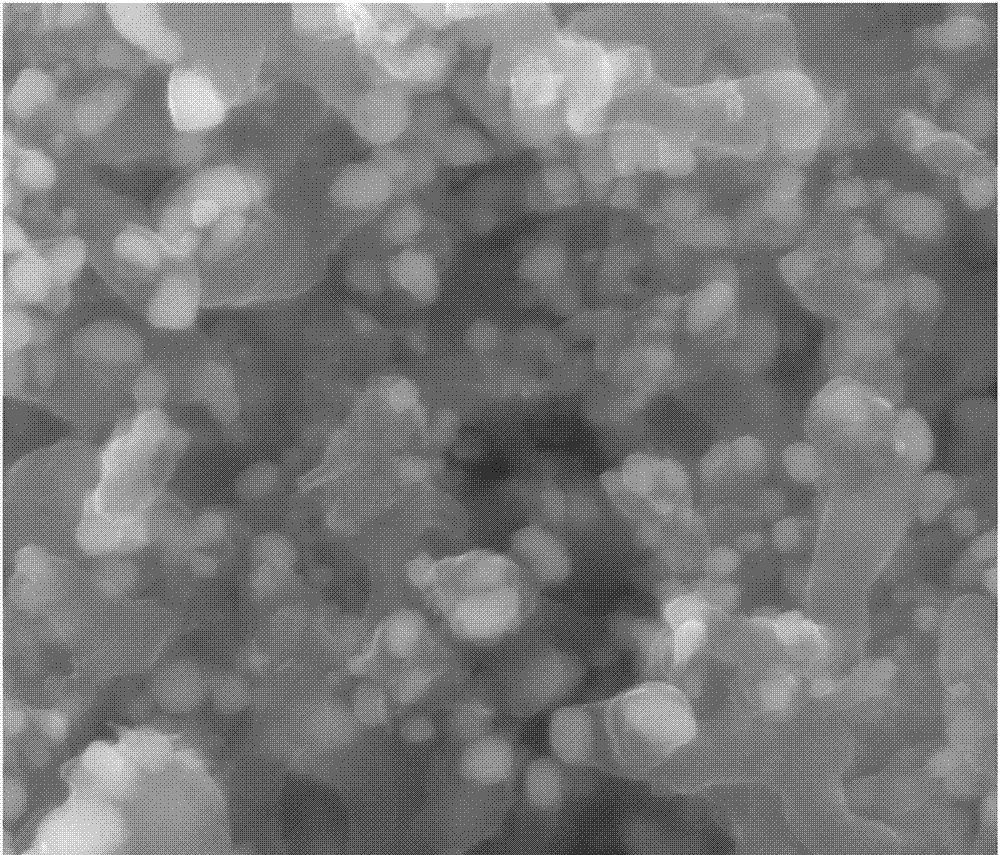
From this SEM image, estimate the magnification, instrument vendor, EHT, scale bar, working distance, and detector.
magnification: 100 K X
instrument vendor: FEI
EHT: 20 kV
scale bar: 500 nm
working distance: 10.4 mm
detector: ETD